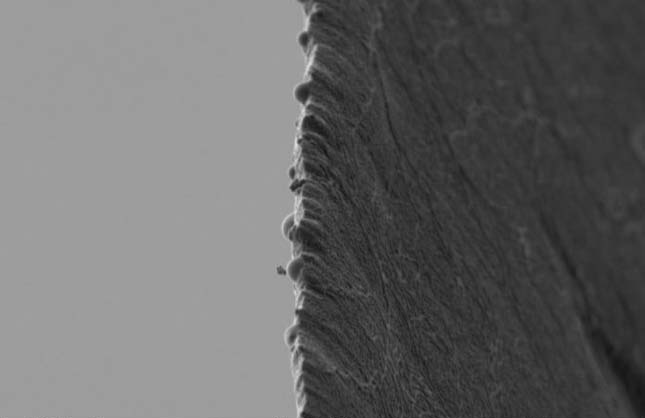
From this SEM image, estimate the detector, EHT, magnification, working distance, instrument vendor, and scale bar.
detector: SE2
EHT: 1 kV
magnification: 10 K X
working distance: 6.1 mm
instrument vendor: Zeiss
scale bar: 1000 nm